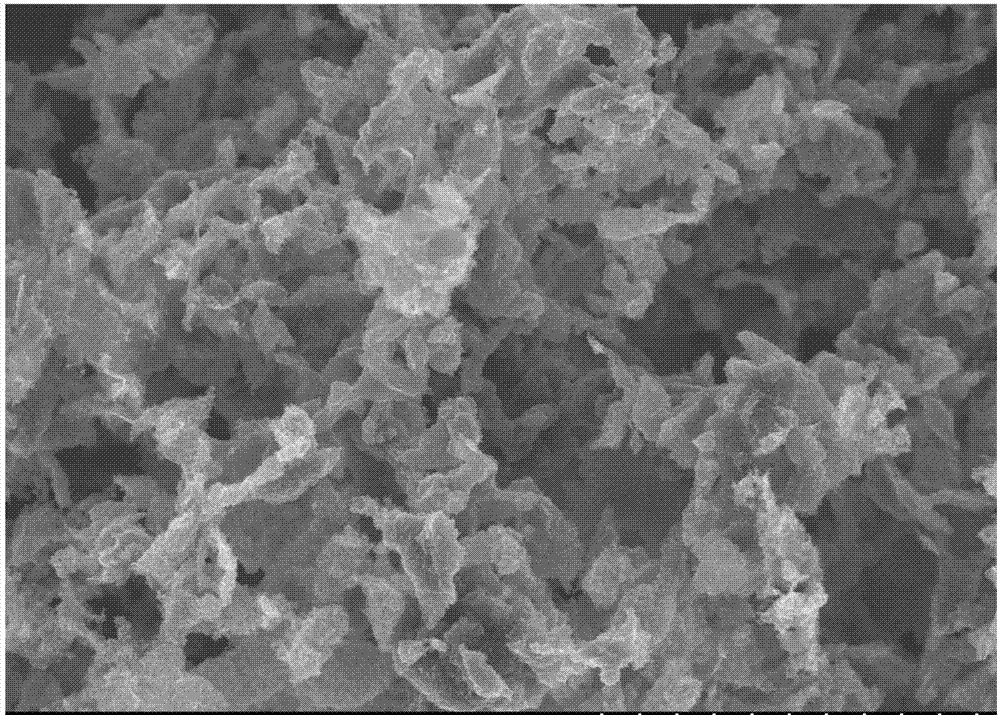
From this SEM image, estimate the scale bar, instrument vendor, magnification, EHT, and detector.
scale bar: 40000 nm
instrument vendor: Hitachi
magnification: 1.2 K X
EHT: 15 kV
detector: SE(U)